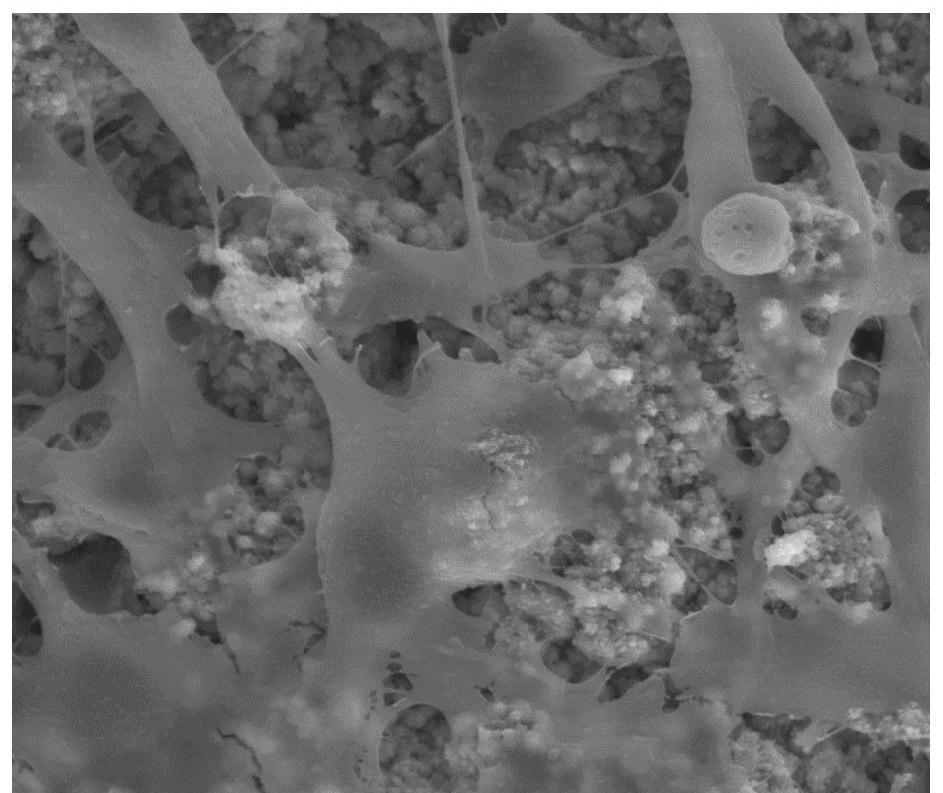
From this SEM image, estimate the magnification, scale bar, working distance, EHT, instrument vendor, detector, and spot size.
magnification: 4 K X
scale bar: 10000 nm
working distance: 10.7 mm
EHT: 15 kV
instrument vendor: FEI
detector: LFD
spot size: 4